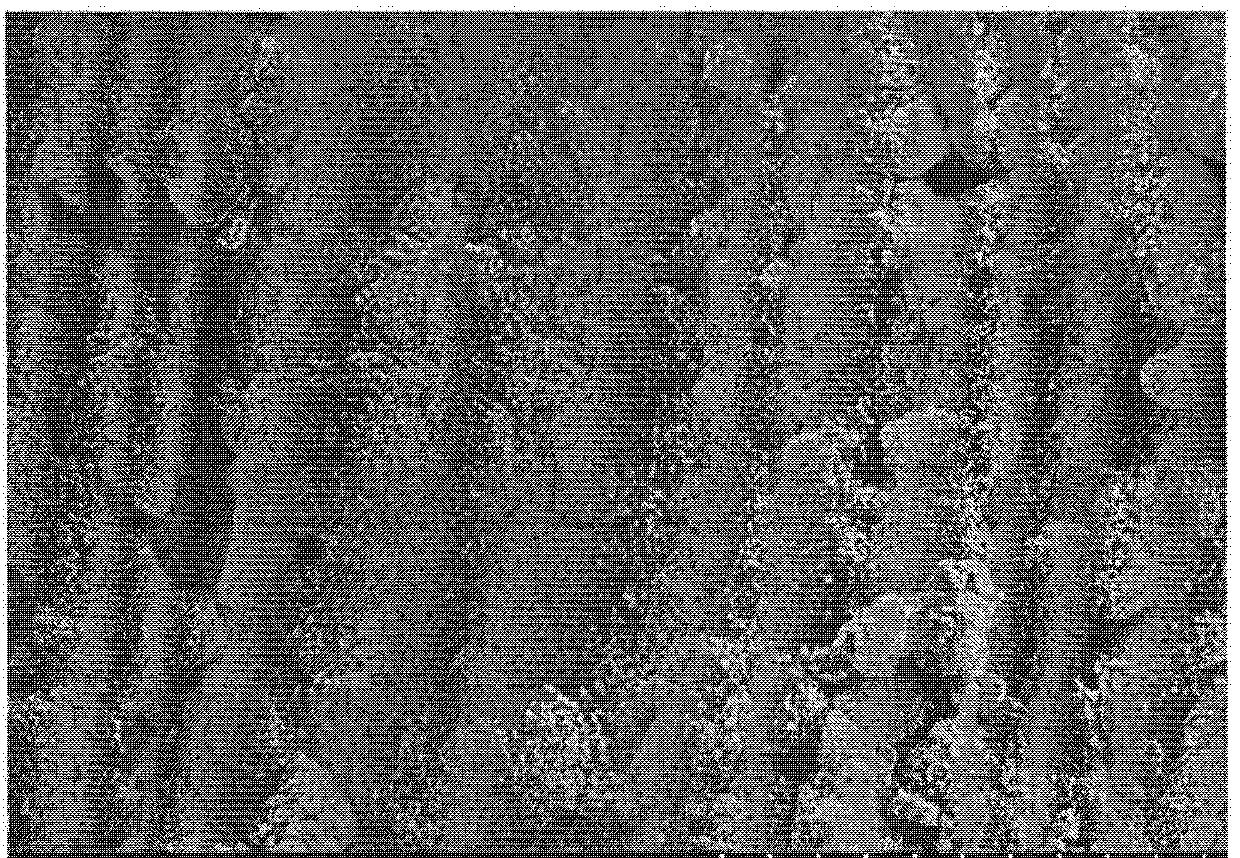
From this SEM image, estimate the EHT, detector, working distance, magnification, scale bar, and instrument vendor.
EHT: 3 kV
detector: SE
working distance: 9.3 mm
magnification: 5 K X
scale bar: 10000 nm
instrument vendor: Hitachi